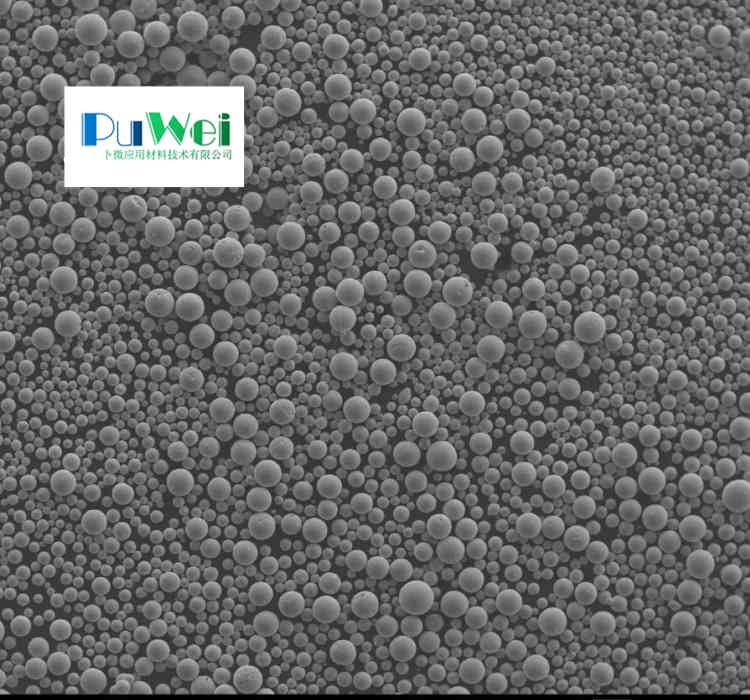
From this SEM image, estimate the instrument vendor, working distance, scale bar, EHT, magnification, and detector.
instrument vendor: JEOL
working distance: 12 mm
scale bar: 100000 nm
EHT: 20 kV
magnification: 0.05 K X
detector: SEI